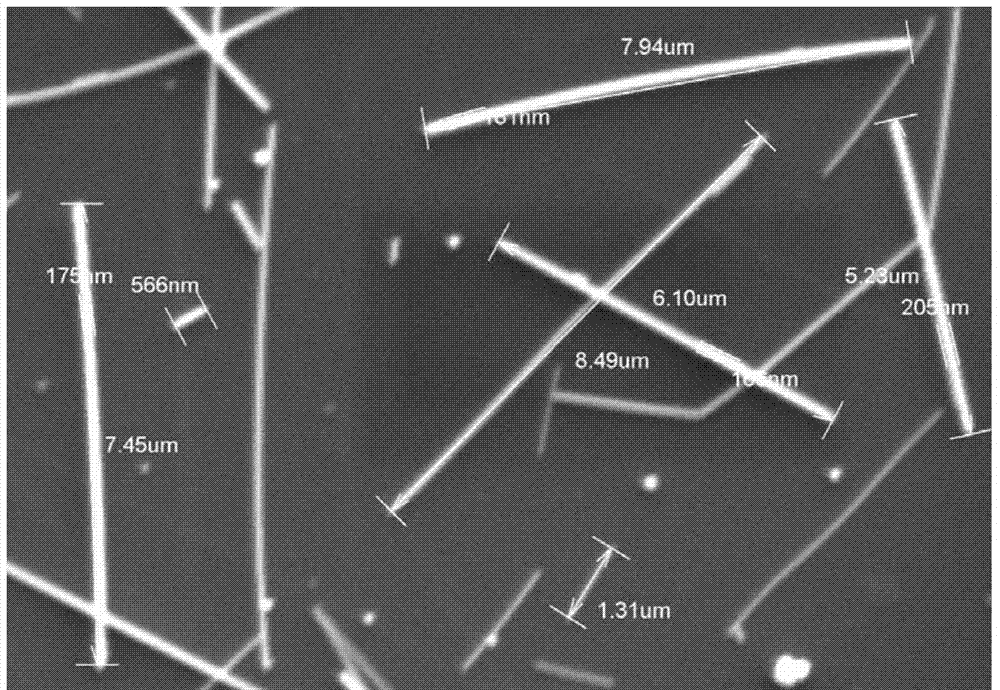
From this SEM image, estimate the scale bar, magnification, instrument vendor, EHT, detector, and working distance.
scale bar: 5000 nm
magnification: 8 K X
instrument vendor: Hitachi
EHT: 10 kV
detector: SE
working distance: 6 mm